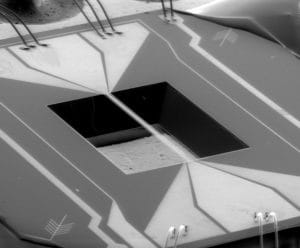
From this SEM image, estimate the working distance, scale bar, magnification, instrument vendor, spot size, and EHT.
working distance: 32.8 mm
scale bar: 1000000 nm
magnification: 0.082 K X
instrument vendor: FEI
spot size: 5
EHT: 15 kV